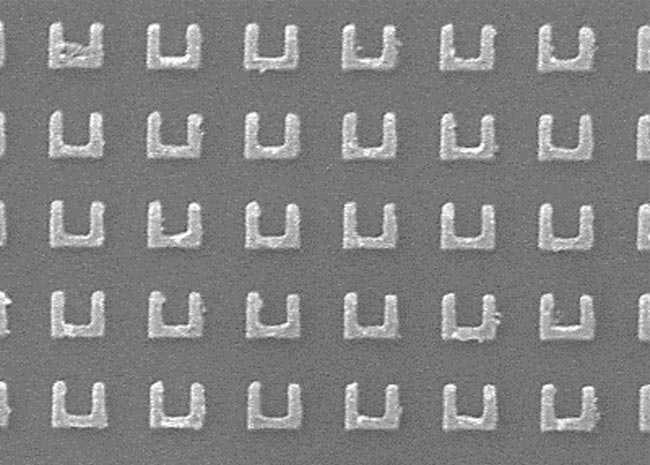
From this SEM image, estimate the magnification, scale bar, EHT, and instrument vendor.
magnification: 30 K X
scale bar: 1000 nm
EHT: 5 kV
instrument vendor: Hitachi